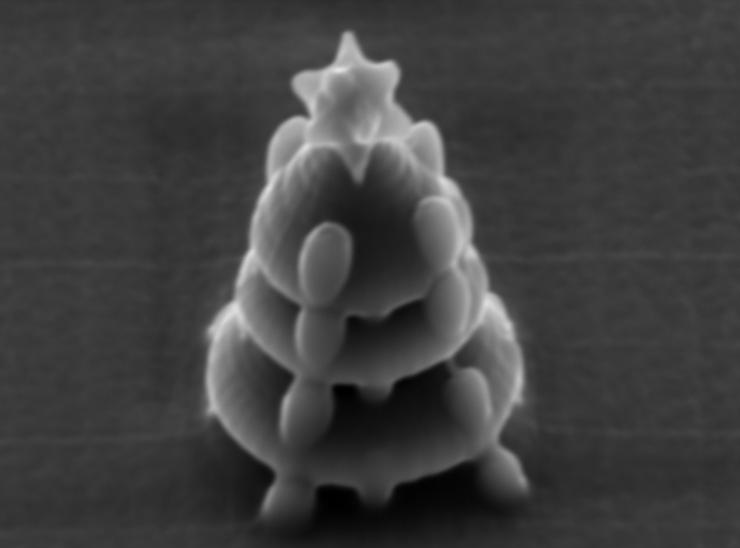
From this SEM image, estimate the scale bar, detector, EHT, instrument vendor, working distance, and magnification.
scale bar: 2000 nm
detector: SED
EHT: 10 kV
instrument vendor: JEOL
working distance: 8.7 mm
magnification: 6.5 K X